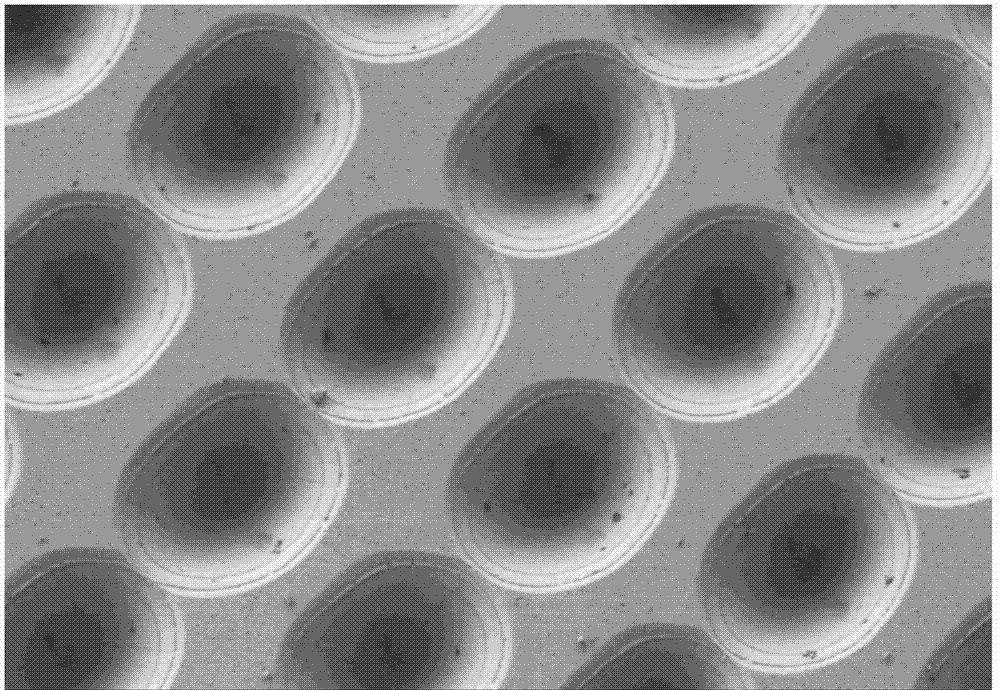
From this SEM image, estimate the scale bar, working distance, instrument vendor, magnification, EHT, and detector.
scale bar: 10000 nm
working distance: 9.3 mm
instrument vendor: Hitachi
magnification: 3 K X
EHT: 1 kV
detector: SE(L)